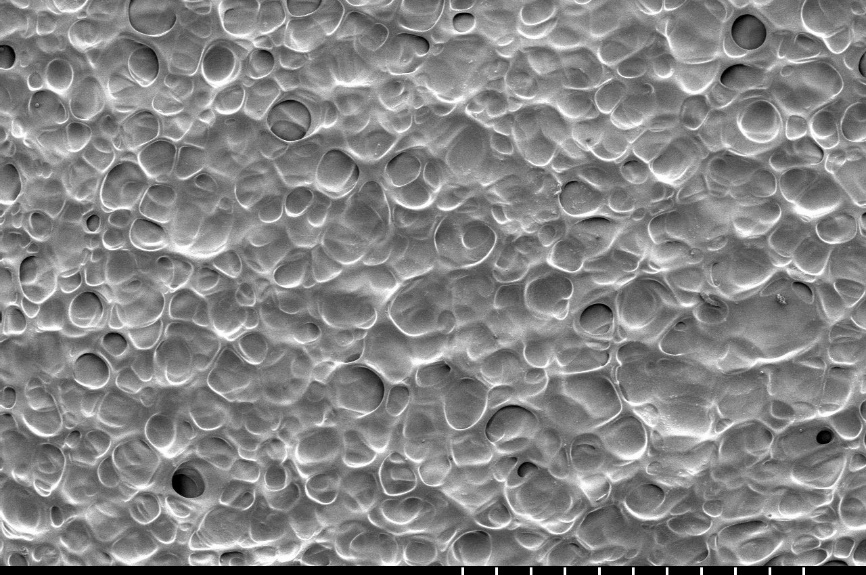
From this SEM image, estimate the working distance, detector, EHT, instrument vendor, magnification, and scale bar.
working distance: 16 mm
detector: SE(M)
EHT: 1.5 kV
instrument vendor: Hitachi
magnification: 0.1 K X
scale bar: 500000 nm